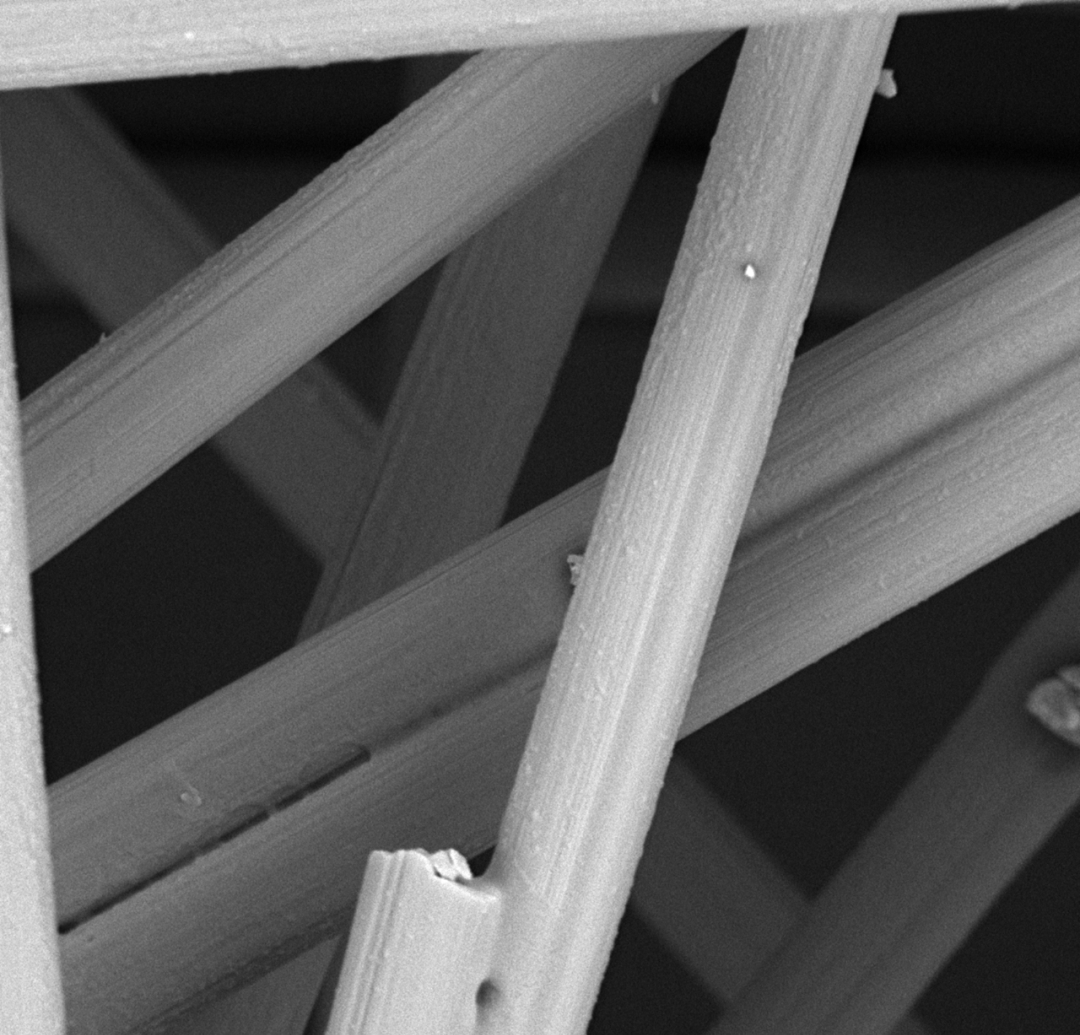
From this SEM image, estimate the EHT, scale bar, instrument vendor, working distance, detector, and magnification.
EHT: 10 kV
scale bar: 10000 nm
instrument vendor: other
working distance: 9.1 mm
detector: BSE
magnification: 5 K X